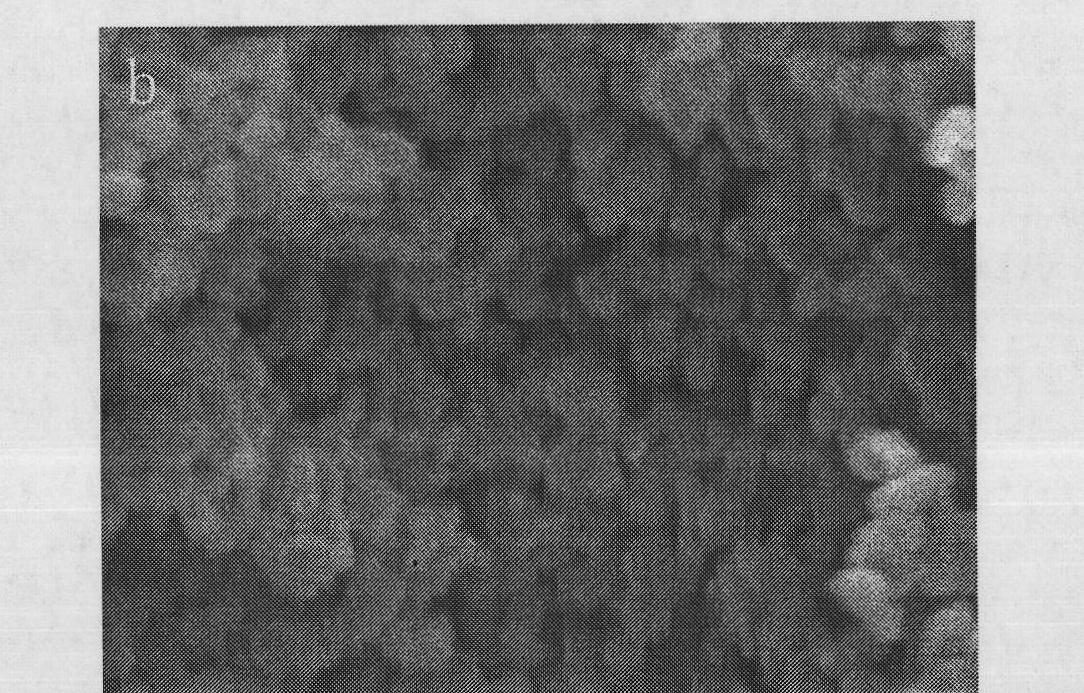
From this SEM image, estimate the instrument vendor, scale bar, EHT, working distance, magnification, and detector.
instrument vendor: JEOL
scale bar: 100 nm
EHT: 5 kV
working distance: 7.7 mm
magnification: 30 K X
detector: SEI